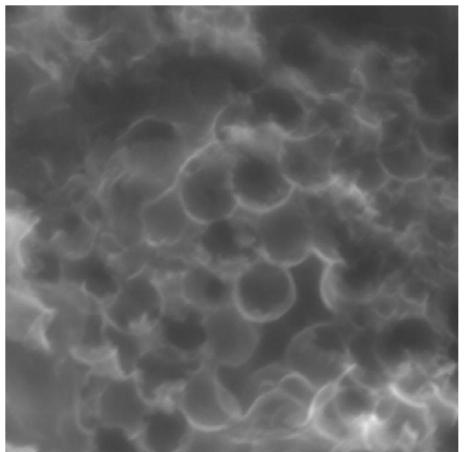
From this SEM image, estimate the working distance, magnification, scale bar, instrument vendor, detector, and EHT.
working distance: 5.02 mm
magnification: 200 K X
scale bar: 200 nm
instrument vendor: Tescan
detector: In-Beam SE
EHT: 10 kV